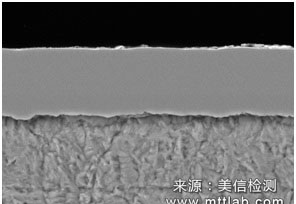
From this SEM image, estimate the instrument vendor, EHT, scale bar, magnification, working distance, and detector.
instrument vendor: Hitachi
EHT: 15 kV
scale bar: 10000 nm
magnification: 5 K X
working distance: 7.7 mm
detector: BSE3D